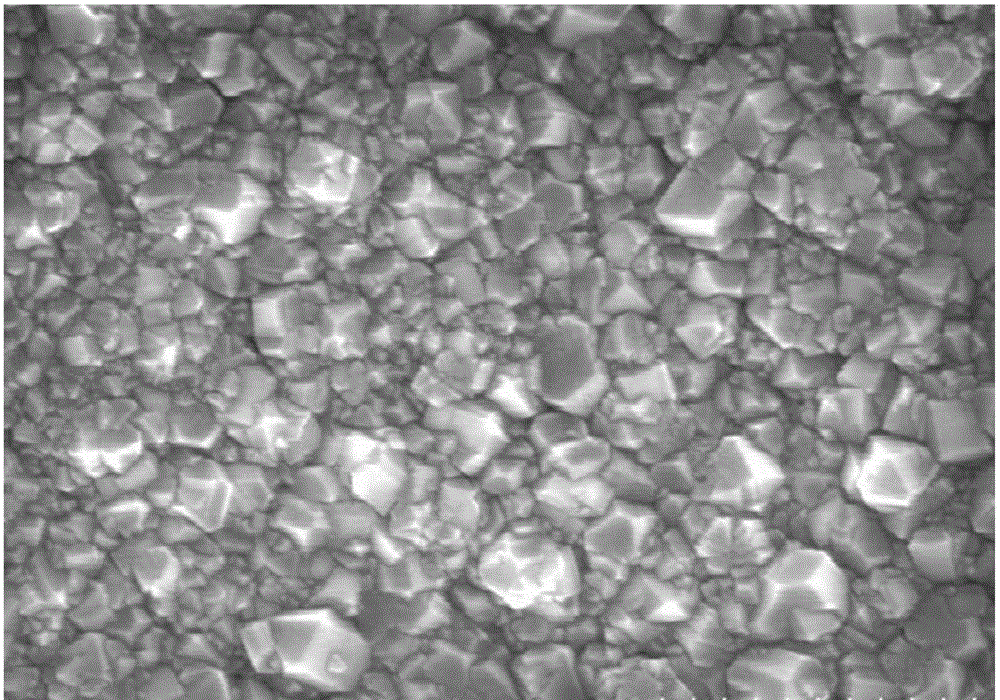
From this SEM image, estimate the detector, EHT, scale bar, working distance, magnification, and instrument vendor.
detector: SE(M)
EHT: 5 kV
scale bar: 1000 nm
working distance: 8.8 mm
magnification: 40 K X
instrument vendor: Hitachi